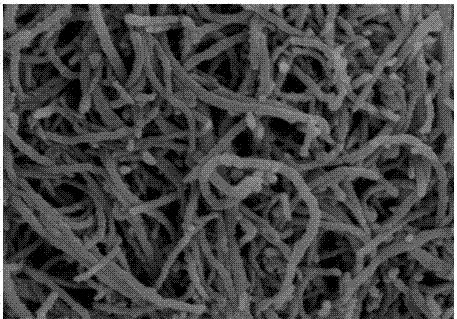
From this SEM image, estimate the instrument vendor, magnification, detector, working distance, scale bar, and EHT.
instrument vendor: Hitachi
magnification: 80 K X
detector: SE(M)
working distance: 11.3 mm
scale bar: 500 nm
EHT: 3 kV